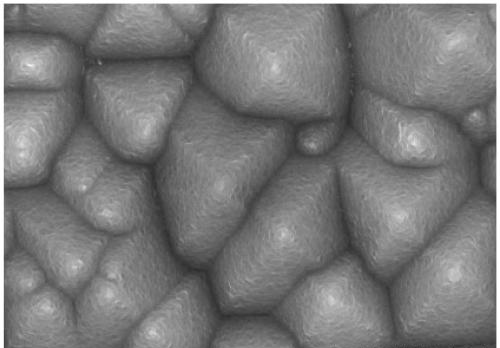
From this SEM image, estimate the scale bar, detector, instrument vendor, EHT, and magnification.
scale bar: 2000 nm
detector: SE(U)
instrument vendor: Hitachi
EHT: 10 kV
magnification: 20 K X